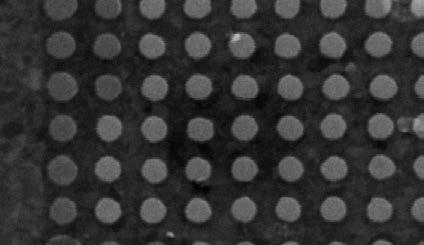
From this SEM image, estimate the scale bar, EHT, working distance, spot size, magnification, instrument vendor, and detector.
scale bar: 500 nm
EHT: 10 kV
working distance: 17.1 mm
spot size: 3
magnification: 65.248 K X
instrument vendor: FEI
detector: SE